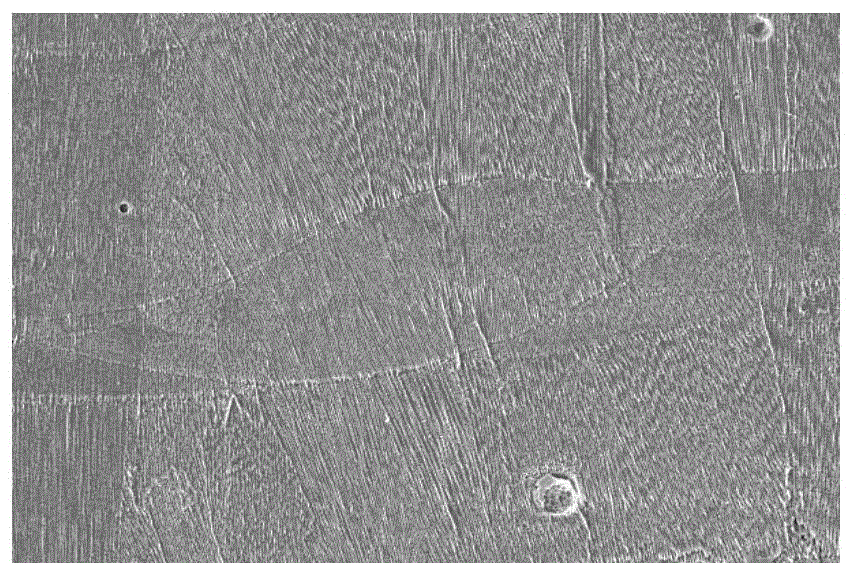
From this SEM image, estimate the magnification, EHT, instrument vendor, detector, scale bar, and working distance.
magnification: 3 K X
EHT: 15 kV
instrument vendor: FEI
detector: ETD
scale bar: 50000 nm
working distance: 11.1 mm